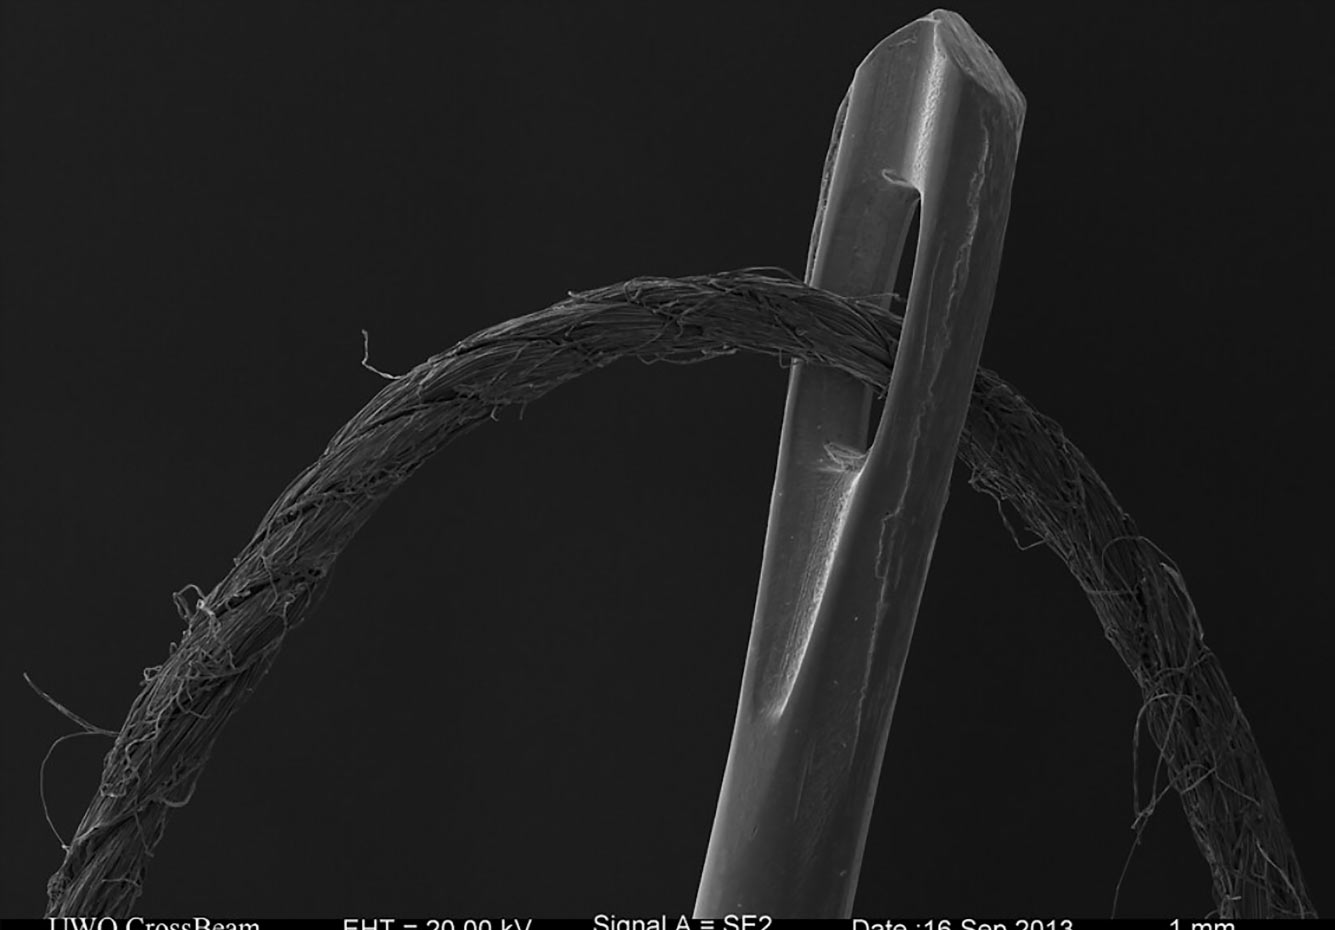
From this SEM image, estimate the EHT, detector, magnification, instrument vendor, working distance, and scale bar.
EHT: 20 kV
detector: SE2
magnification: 0.015 K X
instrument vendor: Zeiss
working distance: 42.7 mm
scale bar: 1000000 nm